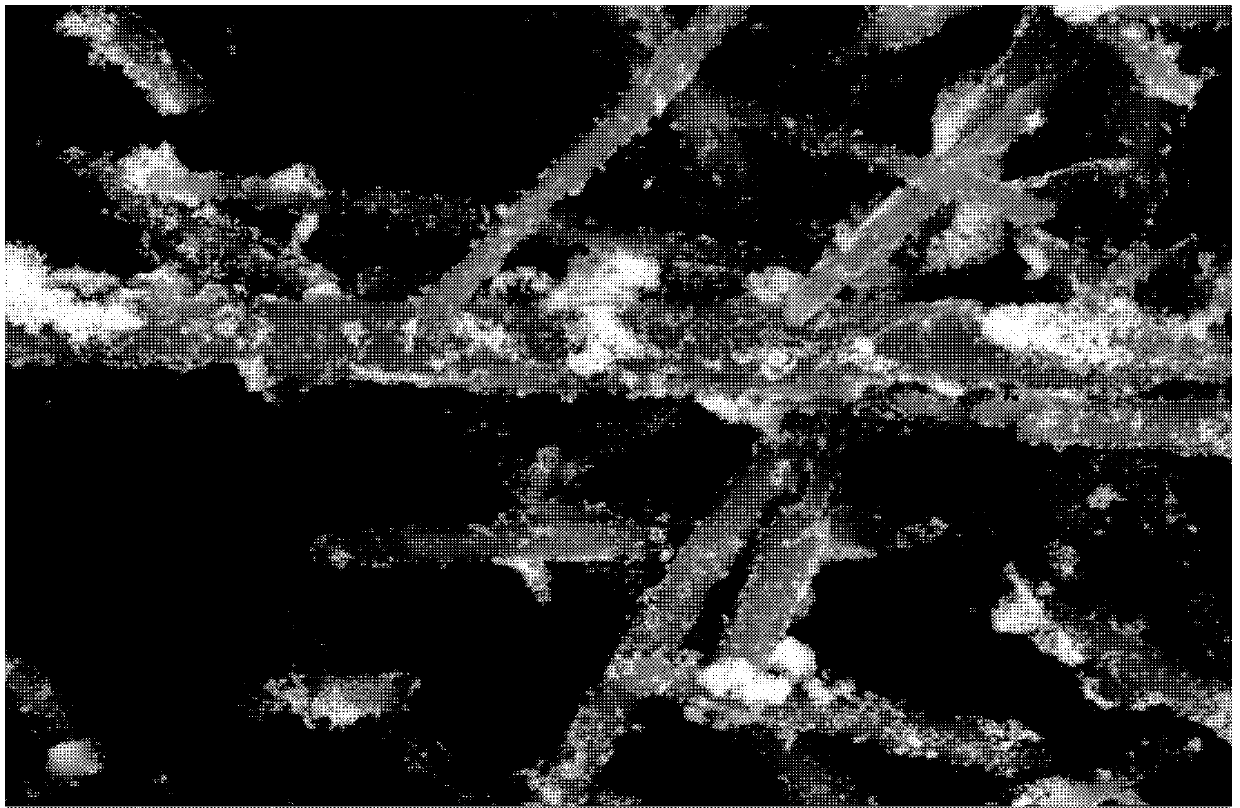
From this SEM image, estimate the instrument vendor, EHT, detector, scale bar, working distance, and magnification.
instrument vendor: FEI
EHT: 20 kV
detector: BSED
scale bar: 10000 nm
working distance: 11.5 mm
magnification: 10 K X